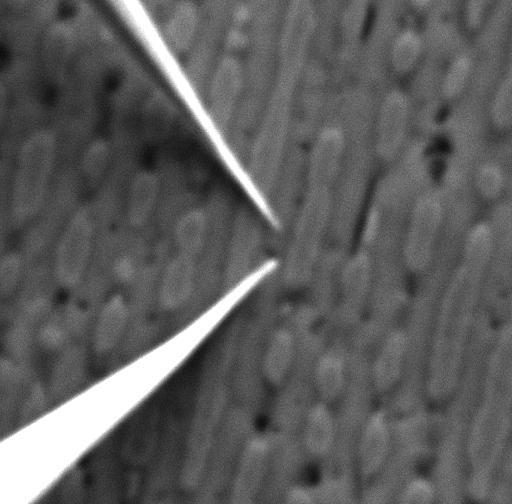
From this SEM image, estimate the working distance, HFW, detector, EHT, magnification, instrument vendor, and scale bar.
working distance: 41.76 mm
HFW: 20.8 µm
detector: SE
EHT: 20 kV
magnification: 6.64 K X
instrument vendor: Tescan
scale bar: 5000 nm